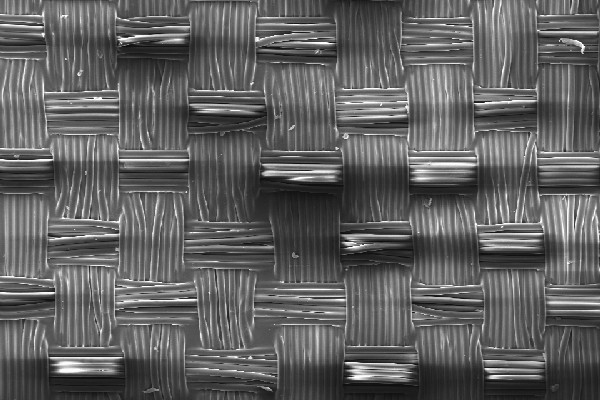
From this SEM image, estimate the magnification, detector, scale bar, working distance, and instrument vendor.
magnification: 0.135 K X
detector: SE1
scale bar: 100000 nm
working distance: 19 mm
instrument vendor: Zeiss